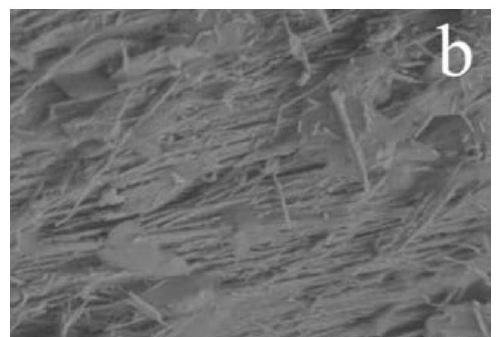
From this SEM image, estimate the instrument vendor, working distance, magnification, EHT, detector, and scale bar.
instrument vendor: Hitachi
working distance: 9.3 mm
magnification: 10 K X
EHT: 3 kV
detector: SE(L)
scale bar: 5000 nm